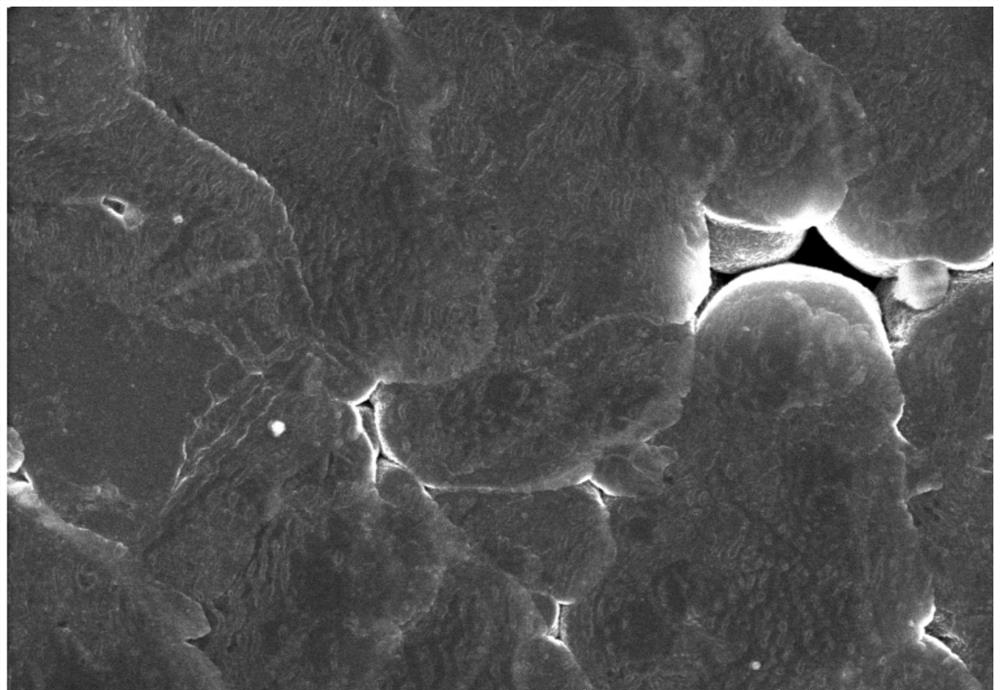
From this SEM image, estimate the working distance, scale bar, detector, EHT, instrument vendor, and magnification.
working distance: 10.9 mm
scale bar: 5000 nm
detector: SE(UL)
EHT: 5 kV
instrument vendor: Hitachi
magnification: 10 K X